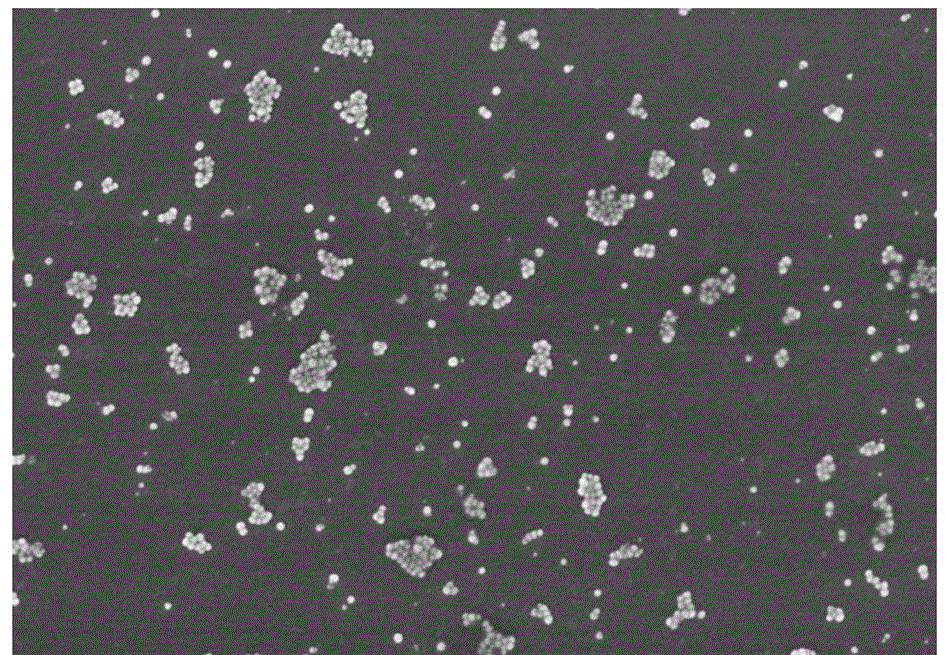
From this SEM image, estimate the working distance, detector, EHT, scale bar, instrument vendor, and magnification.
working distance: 8.7 mm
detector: SE(U,LA0)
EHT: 3 kV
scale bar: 1000 nm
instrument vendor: Hitachi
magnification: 30 K X